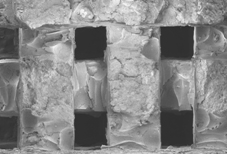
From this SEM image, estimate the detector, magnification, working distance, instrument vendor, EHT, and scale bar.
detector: SE(M)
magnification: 0.03 K X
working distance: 12.4 mm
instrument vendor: Hitachi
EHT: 5 kV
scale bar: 1000000 nm